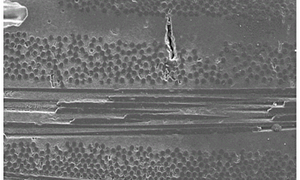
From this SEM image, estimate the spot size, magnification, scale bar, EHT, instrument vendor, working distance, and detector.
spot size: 5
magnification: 0.5 K X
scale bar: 200000 nm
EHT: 20 kV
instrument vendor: FEI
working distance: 11.3 mm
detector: ETD